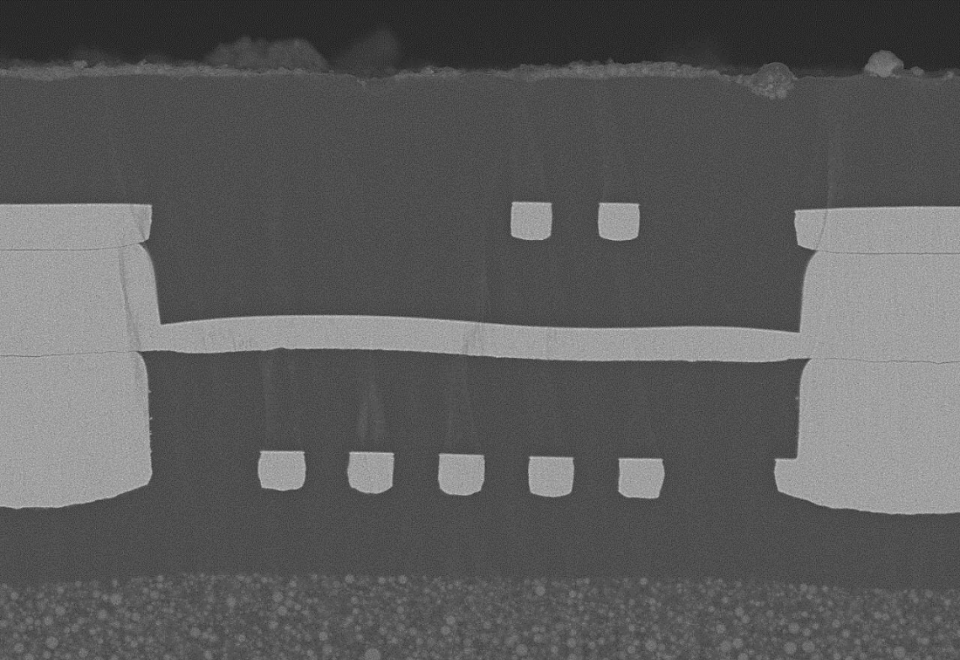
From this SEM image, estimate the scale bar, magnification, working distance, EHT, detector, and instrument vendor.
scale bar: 30000 nm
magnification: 1.5 K X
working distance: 8 mm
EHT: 10 kV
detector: LA100(U)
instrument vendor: Hitachi